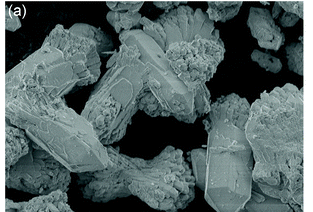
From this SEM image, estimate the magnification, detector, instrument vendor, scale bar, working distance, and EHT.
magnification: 0.55 K X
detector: LED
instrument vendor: JEOL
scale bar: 10000 nm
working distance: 9.9 mm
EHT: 5 kV